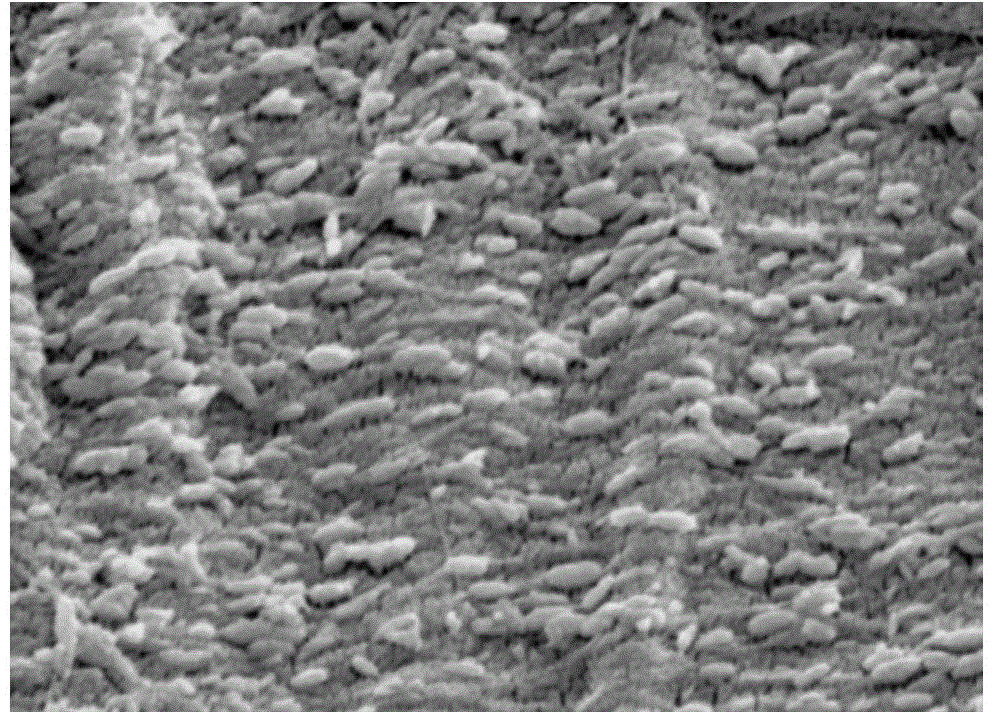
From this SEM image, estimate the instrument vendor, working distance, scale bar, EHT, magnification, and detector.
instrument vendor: JEOL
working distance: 10.1 mm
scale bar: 100 nm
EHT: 5 kV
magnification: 30 K X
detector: SEI